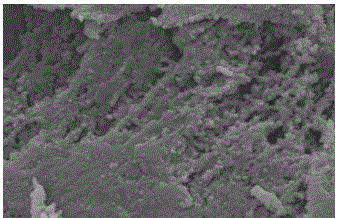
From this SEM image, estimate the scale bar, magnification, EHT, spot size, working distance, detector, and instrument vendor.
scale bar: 500 nm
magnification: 40 K X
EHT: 20 kV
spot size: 3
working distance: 4.8 mm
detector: SE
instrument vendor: FEI